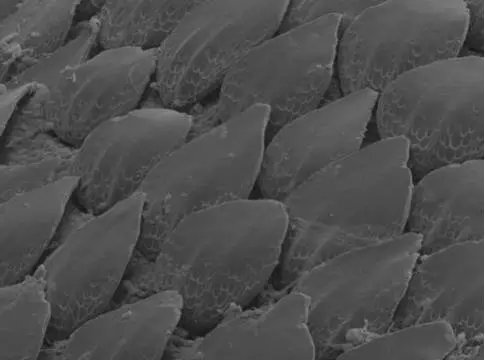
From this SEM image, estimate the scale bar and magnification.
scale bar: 20000 nm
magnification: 0.187 K X